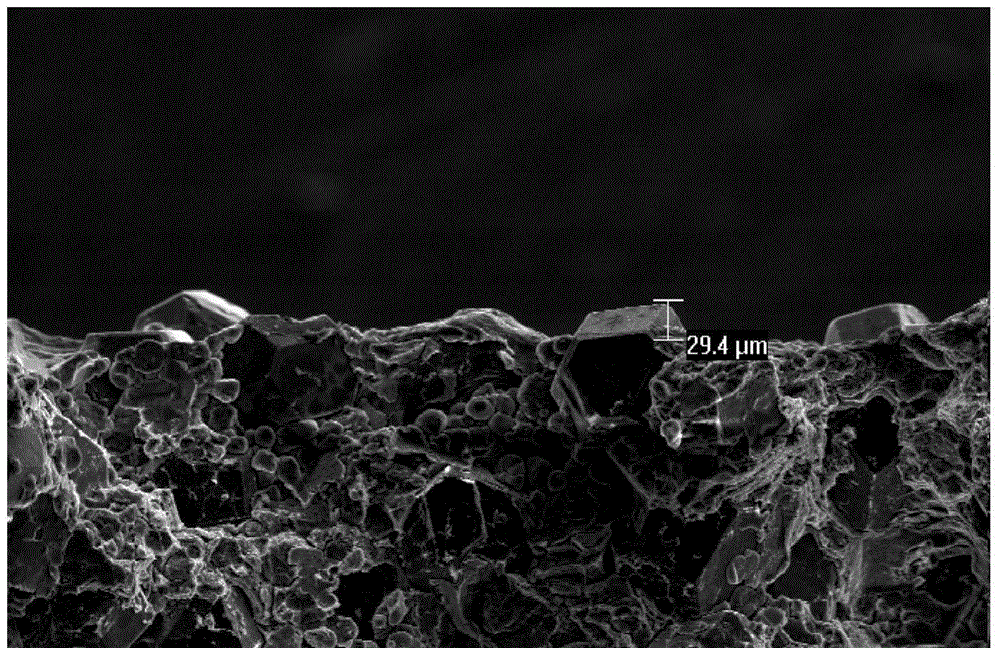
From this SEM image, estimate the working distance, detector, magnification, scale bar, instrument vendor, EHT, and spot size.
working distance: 11.3 mm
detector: SE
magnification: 0.35 K X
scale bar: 200000 nm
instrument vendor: FEI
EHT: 20 kV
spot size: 4